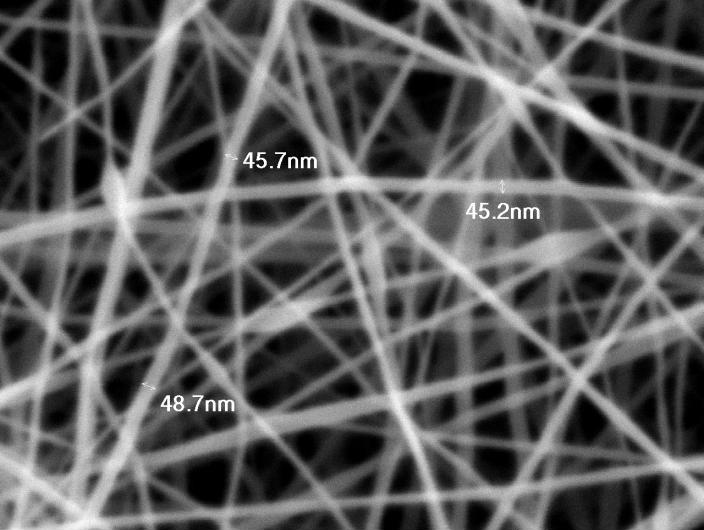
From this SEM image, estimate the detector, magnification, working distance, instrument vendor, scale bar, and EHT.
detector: SEI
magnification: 50 K X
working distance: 9.9 mm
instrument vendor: JEOL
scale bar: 100 nm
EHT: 5 kV